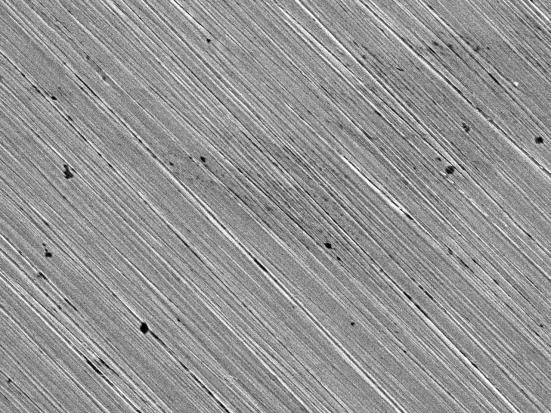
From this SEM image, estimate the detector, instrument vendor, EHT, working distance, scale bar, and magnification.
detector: SEI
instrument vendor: JEOL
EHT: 20 kV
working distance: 6.1 mm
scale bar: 10000 nm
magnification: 0.8 K X